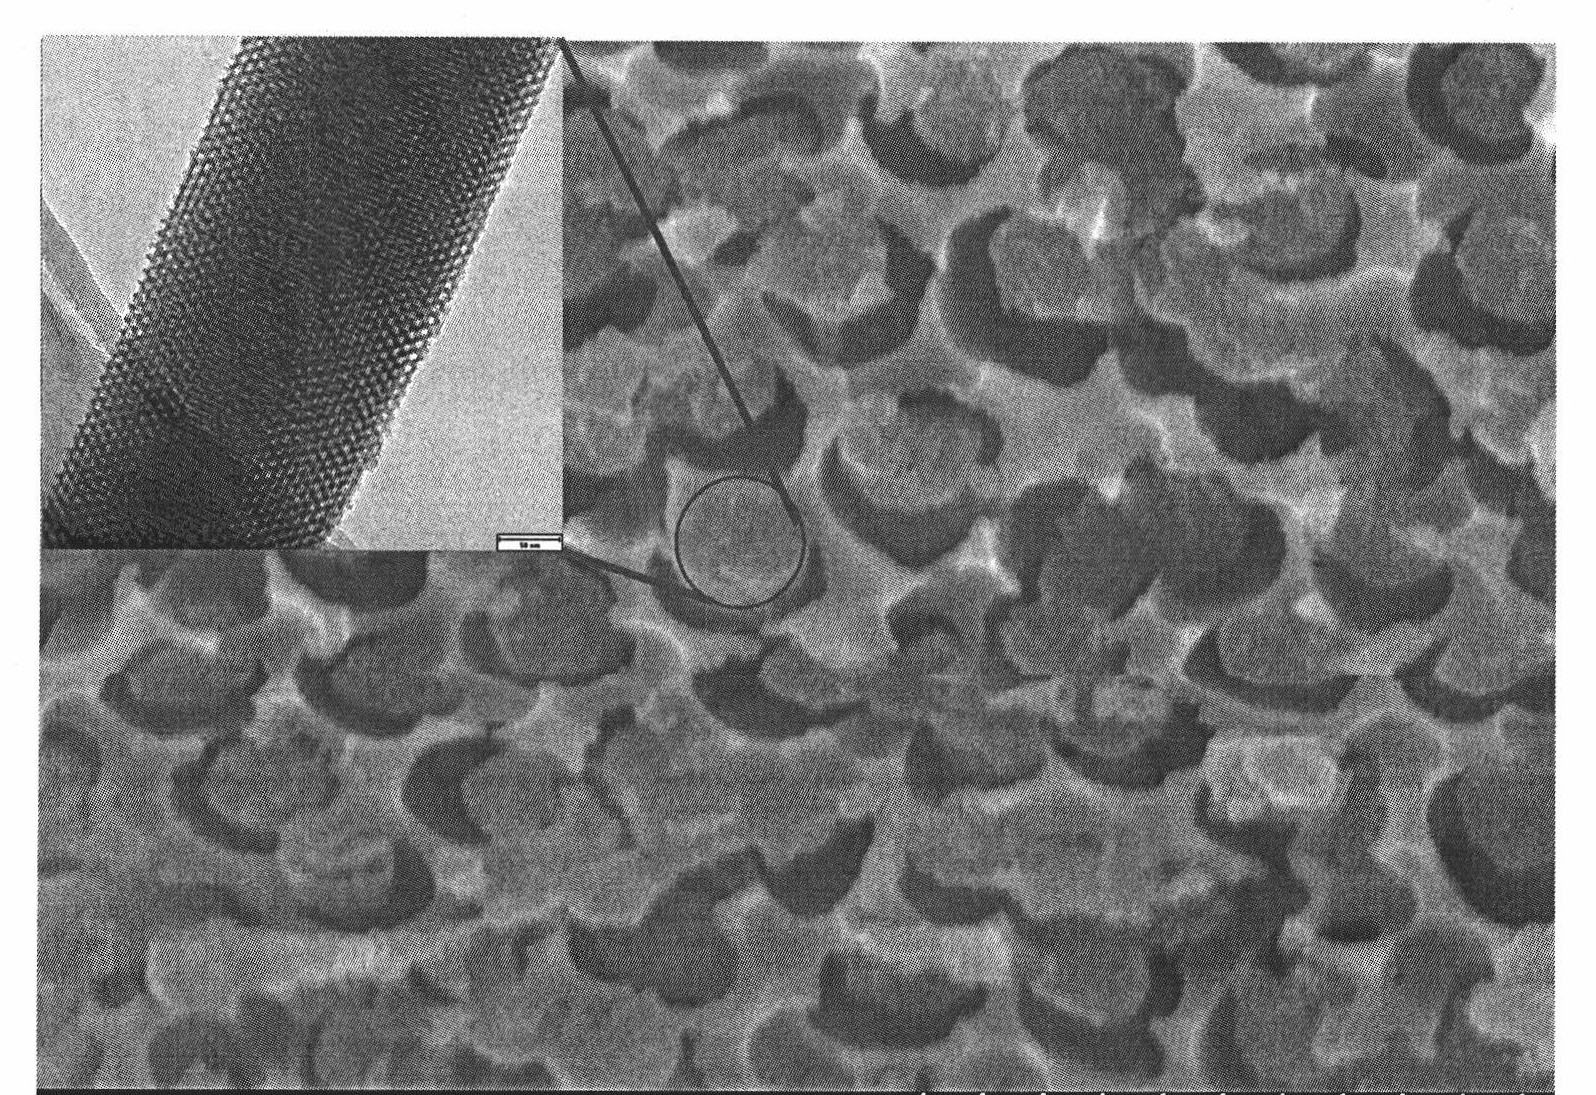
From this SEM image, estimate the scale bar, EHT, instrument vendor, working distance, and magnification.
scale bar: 1000 nm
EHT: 20 kV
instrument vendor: Hitachi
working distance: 11.5 mm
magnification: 50 K X